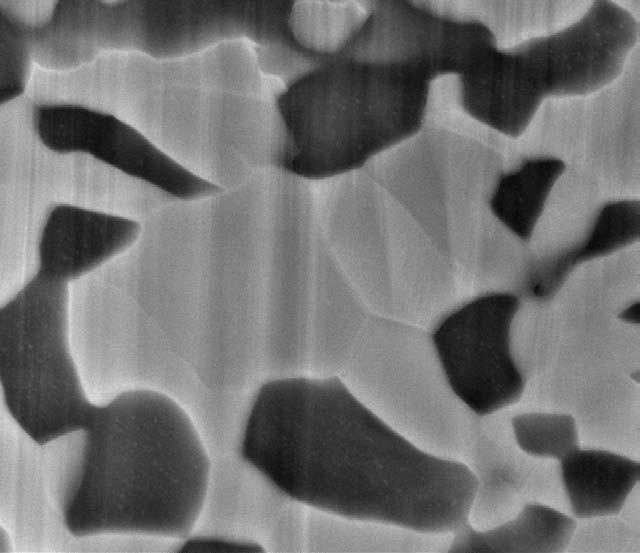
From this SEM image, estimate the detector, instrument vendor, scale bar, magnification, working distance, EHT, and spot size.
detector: ETD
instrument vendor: FEI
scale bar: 2000 nm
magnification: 29.939 K X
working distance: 10.5 mm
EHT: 10 kV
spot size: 4.5